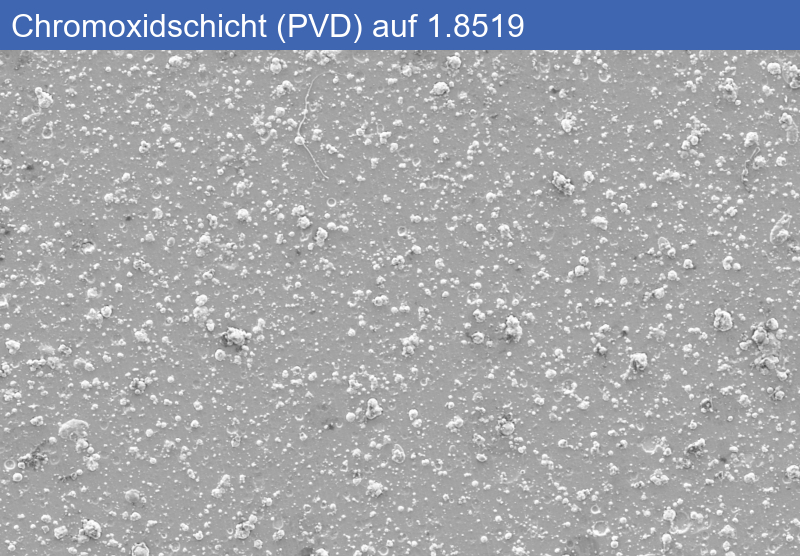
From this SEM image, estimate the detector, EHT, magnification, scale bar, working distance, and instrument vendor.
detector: SE2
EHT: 5 kV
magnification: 0.5 K X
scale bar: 10000 nm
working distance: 11.4 mm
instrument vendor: Zeiss